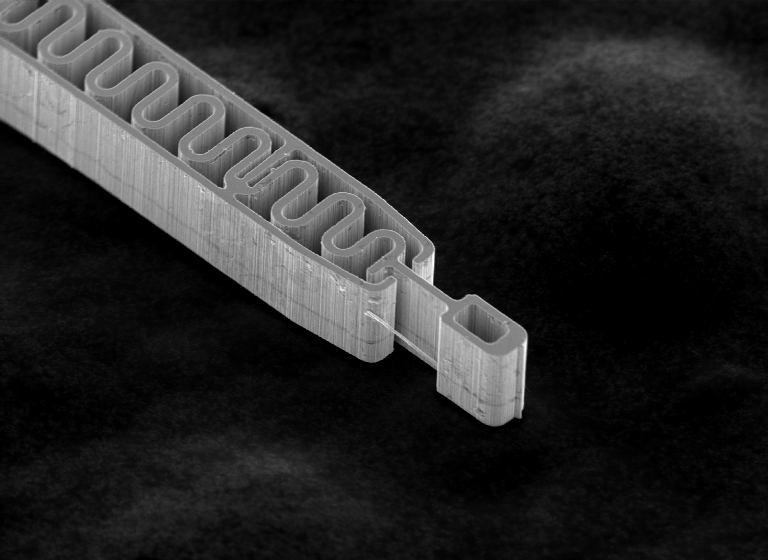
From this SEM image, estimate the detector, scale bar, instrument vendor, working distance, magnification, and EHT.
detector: SEI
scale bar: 50000 nm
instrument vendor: JEOL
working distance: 9.9 mm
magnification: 0.6 K X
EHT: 20 kV